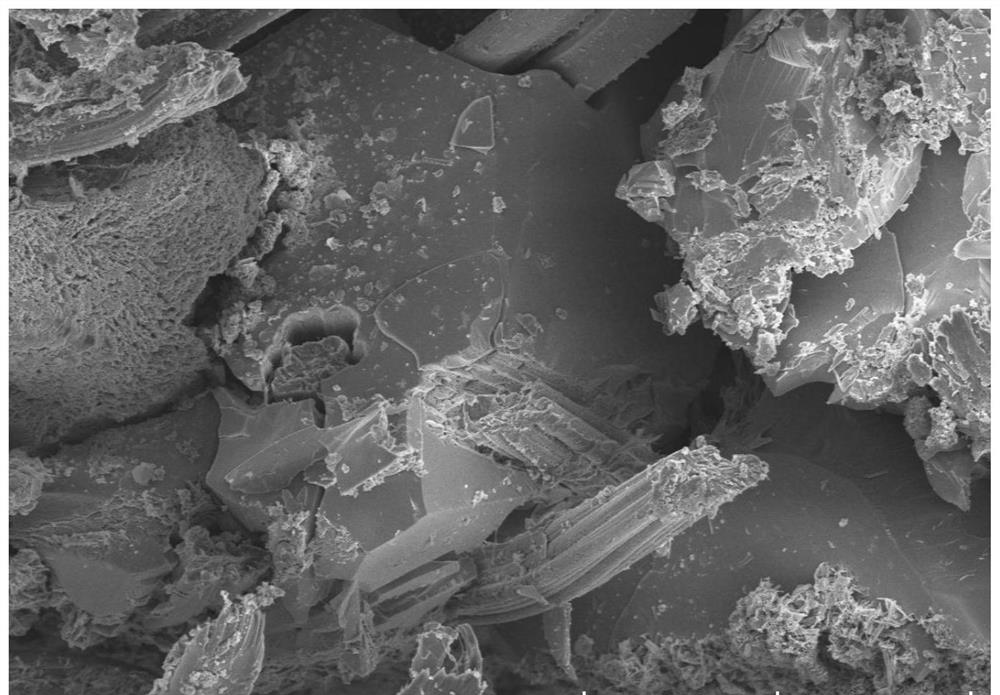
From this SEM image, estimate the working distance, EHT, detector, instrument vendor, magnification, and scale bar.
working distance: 8.8 mm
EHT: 5 kV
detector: SE(UL)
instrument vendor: Hitachi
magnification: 1 K X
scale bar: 50000 nm